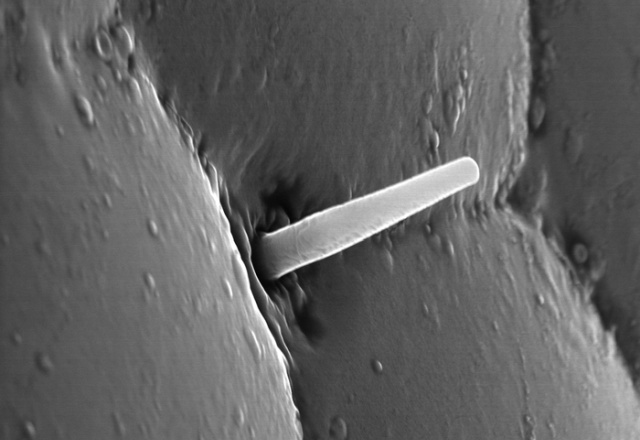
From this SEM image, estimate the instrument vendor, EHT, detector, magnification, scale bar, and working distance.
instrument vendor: Hitachi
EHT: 30 kV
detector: SE(UL)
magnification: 8 K X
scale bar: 5000 nm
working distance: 8 mm